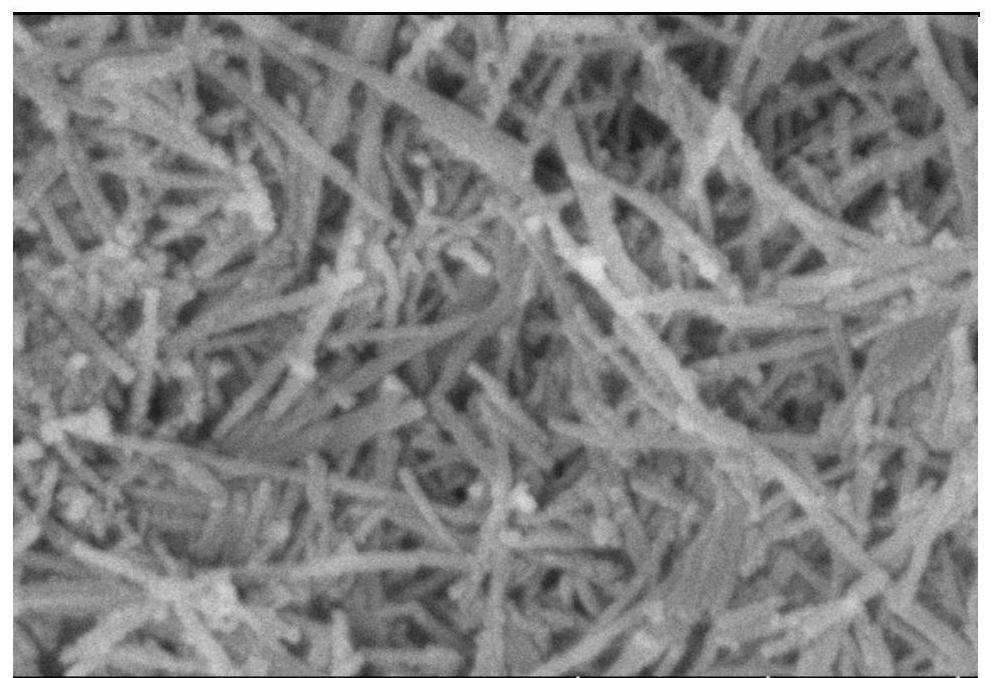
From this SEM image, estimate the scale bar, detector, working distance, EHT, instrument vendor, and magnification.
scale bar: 500 nm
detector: SE(UL)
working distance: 8.6 mm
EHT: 5 kV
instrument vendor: Hitachi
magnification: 100 K X